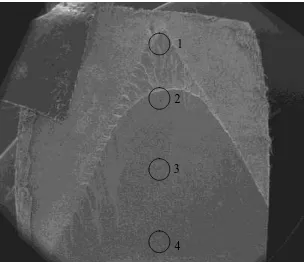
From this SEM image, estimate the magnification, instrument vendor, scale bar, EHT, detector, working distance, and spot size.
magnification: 0.019 K X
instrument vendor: FEI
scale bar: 5000000 nm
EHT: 10 kV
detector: ETD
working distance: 31.7 mm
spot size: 3